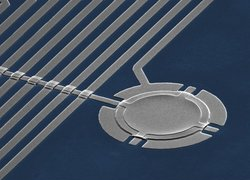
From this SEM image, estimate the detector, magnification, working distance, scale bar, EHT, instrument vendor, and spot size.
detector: SE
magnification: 2.5 K X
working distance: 23.3 mm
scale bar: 10000 nm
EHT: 30 kV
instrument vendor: FEI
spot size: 3